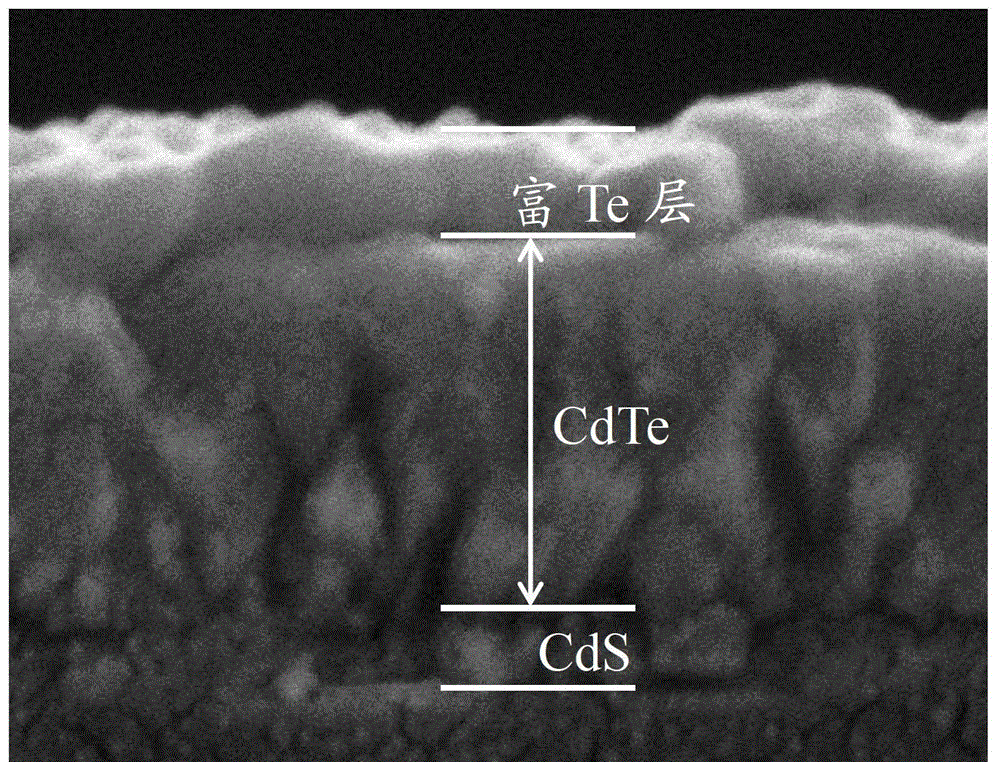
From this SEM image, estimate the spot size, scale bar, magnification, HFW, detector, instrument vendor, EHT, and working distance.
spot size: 2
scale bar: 1000 nm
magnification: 40 K X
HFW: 3.73 µm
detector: ETD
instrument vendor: FEI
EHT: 10 kV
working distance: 4.9 mm